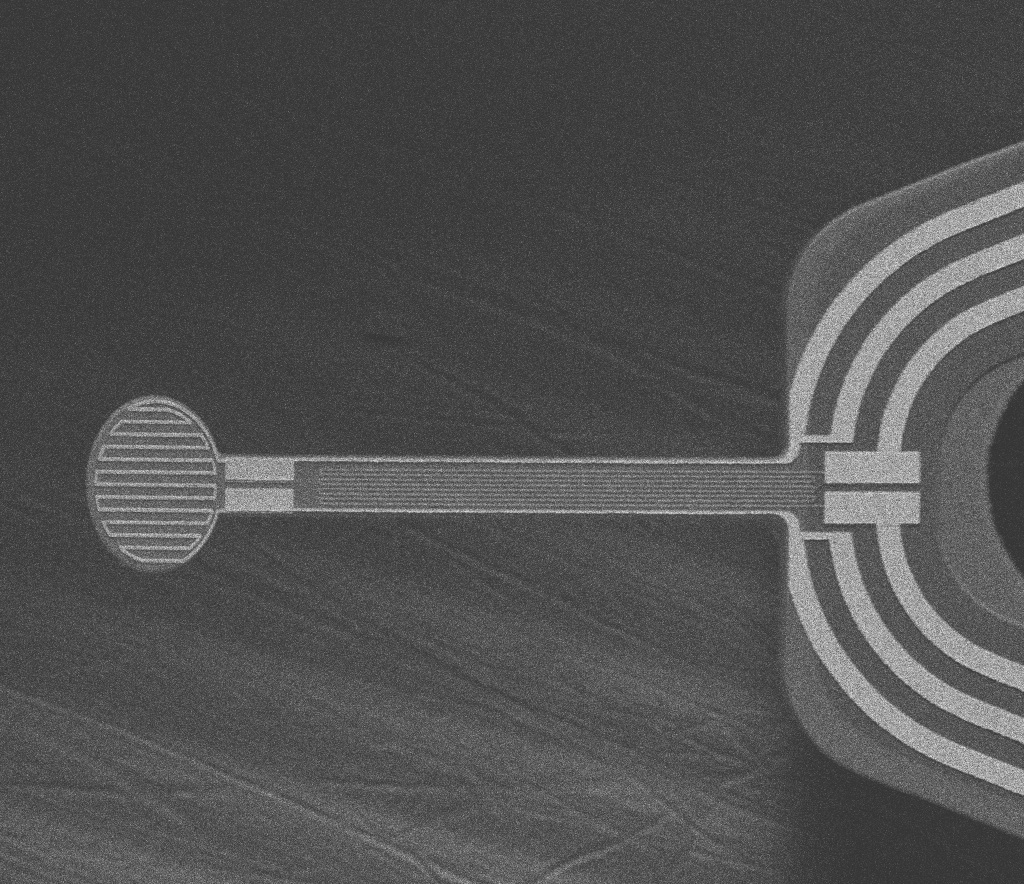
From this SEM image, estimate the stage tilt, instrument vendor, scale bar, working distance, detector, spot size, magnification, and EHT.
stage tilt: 0°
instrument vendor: FEI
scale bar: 200000 nm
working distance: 30 mm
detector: ETD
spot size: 3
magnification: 0.2 K X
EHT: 15 kV